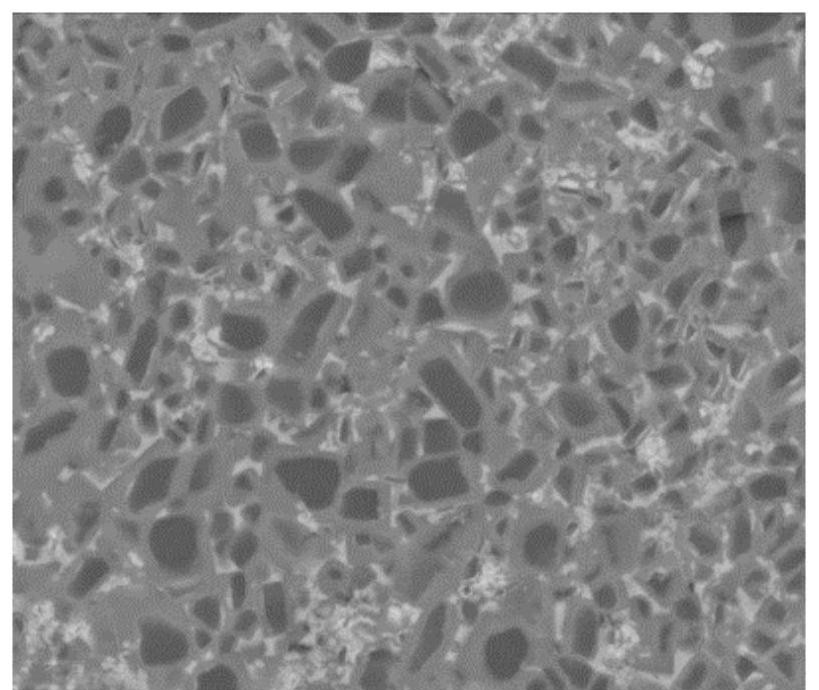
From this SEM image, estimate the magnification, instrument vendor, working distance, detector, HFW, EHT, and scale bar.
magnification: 10 K X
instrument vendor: FEI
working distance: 10.9 mm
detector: BSED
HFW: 29.8 µm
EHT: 20 kV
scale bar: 10000 nm